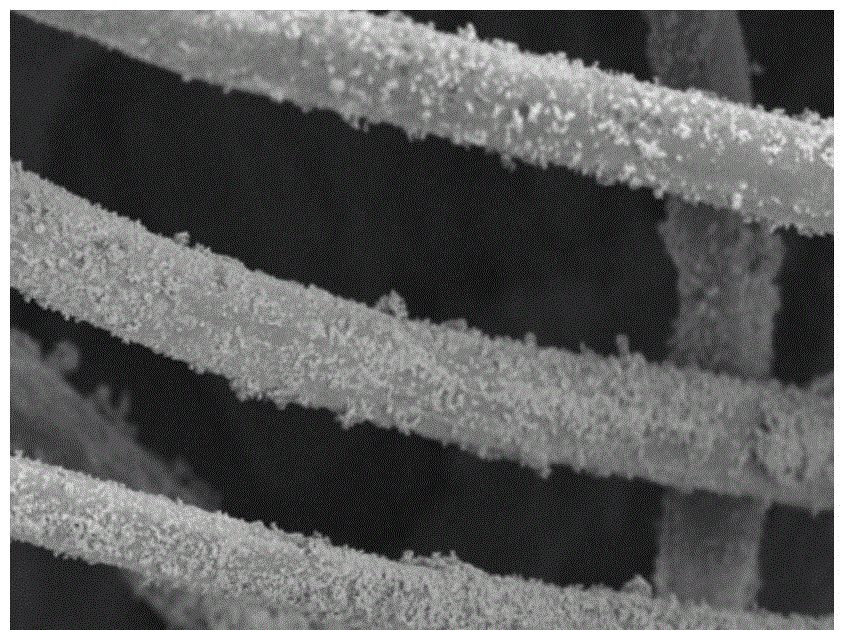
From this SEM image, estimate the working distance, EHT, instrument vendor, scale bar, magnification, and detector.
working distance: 12.2 mm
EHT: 5 kV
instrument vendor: Hitachi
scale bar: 50000 nm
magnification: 1 K X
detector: SE(UL)